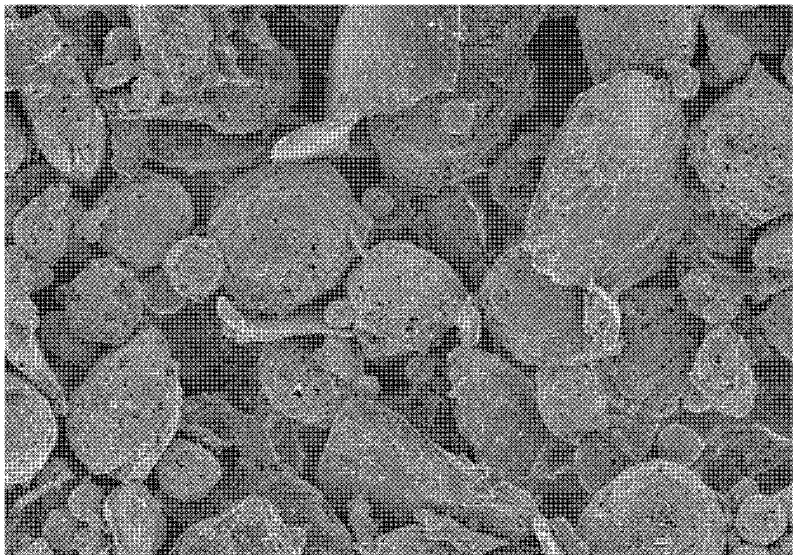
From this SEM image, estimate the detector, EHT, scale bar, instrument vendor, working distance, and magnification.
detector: SE(M)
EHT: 3 kV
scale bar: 50000 nm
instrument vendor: Hitachi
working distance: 8.5 mm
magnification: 1 K X